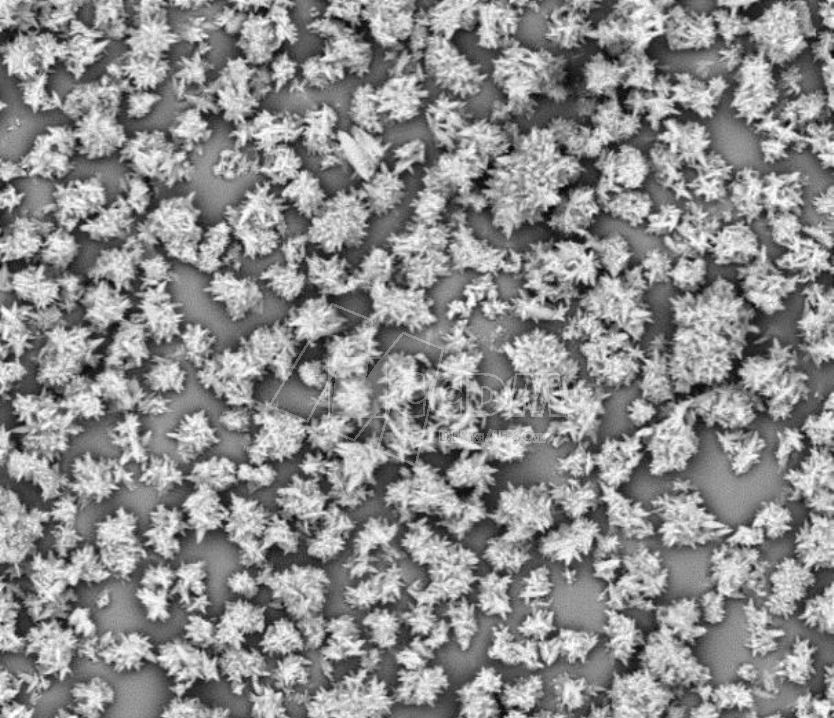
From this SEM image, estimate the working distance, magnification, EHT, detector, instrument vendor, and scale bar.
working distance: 12 mm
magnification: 3 K X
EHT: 20 kV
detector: BSED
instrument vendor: FEI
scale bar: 40000 nm